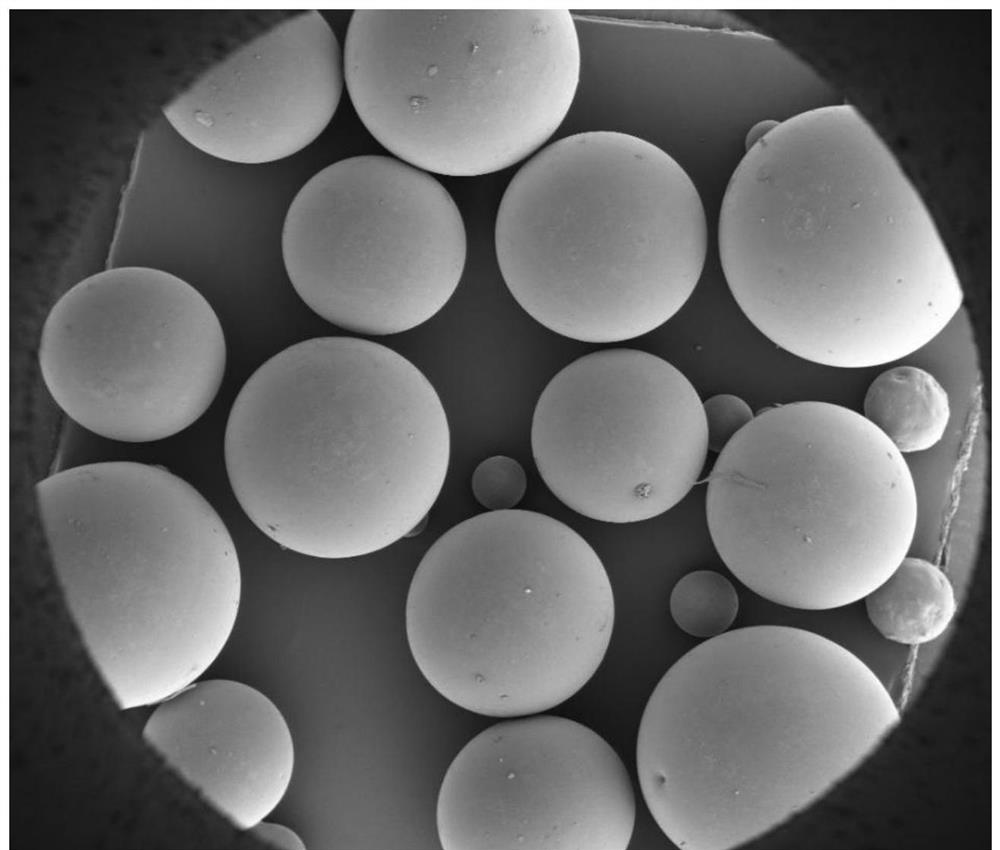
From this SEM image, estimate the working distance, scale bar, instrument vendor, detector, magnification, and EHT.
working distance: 6.6 mm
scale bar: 1000000 nm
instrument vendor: FEI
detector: ETD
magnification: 0.036 K X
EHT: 3 kV